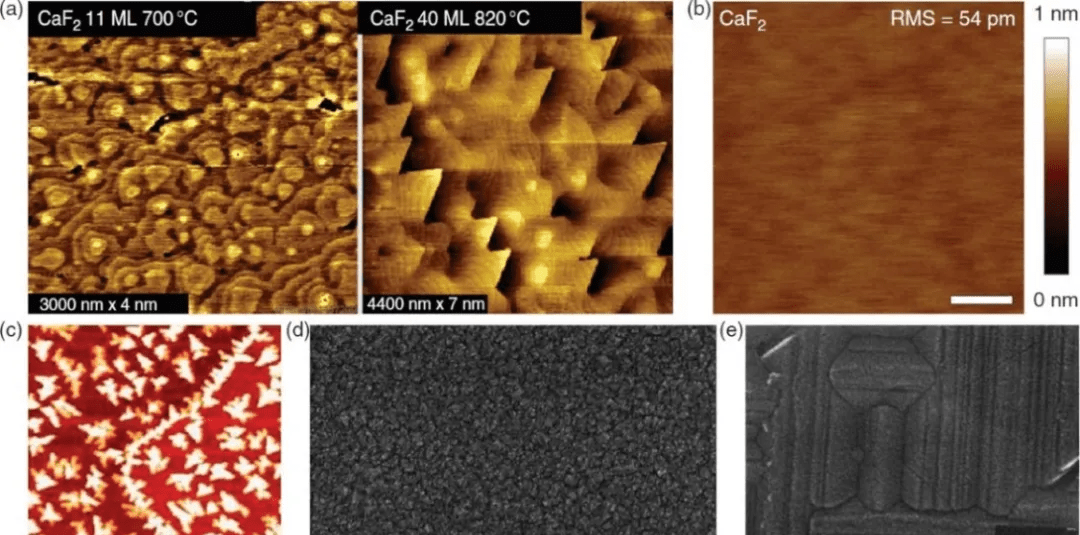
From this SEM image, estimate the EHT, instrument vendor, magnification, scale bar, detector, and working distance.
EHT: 10 kV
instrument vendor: Hitachi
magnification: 80 K X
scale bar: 500 nm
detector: SE(M)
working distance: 3.7 mm